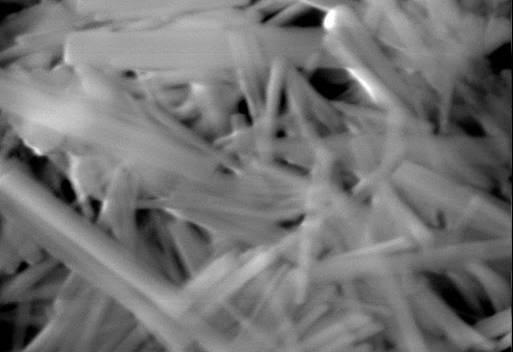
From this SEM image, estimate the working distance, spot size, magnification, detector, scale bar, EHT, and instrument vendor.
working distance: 11.6 mm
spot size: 5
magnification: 10 K X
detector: ETD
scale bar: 5000 nm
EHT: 30 kV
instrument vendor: FEI